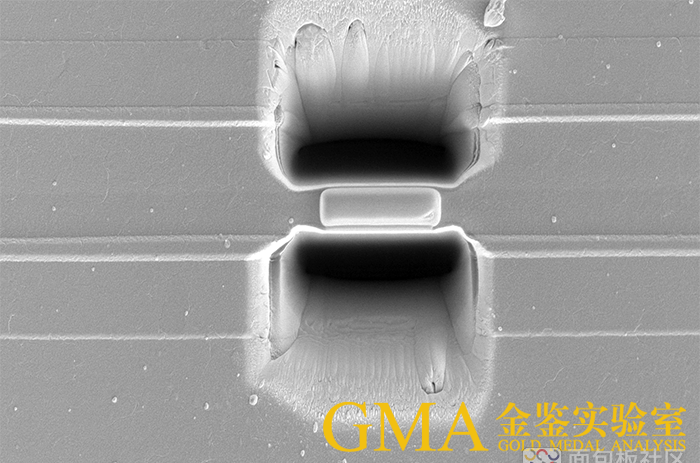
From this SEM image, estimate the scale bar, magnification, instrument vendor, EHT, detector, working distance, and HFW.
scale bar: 20000 nm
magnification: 2.5 K X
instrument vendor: FEI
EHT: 5 kV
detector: ETD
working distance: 7 mm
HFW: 50.8 µm